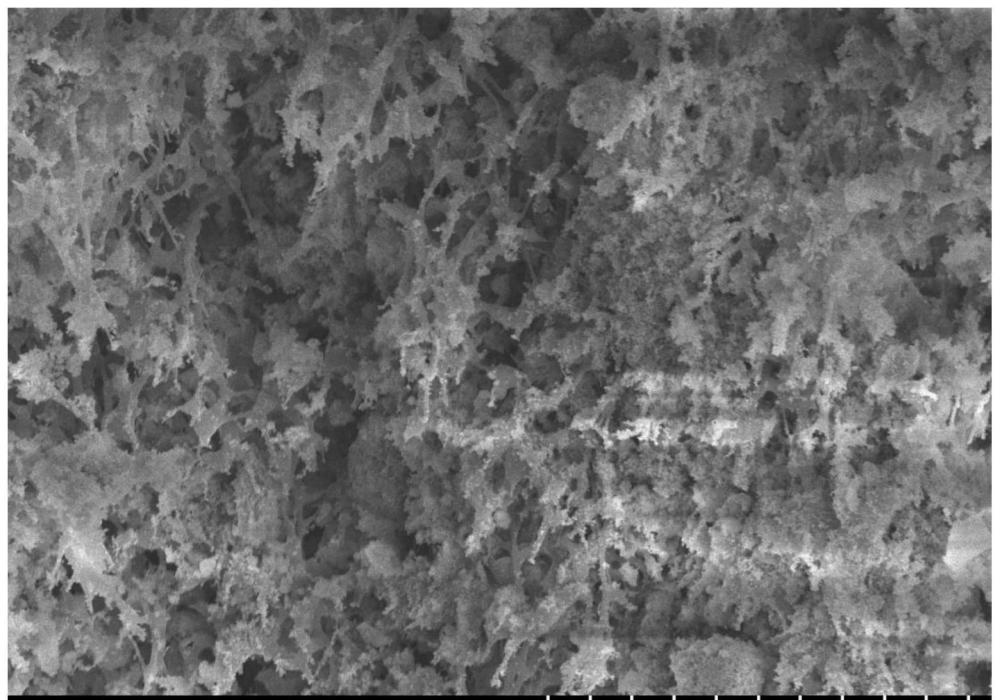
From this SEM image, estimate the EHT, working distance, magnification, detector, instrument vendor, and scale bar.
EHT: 10 kV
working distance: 13.1 mm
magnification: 1.81 K X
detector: SE(UL)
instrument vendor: Hitachi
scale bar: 30000 nm